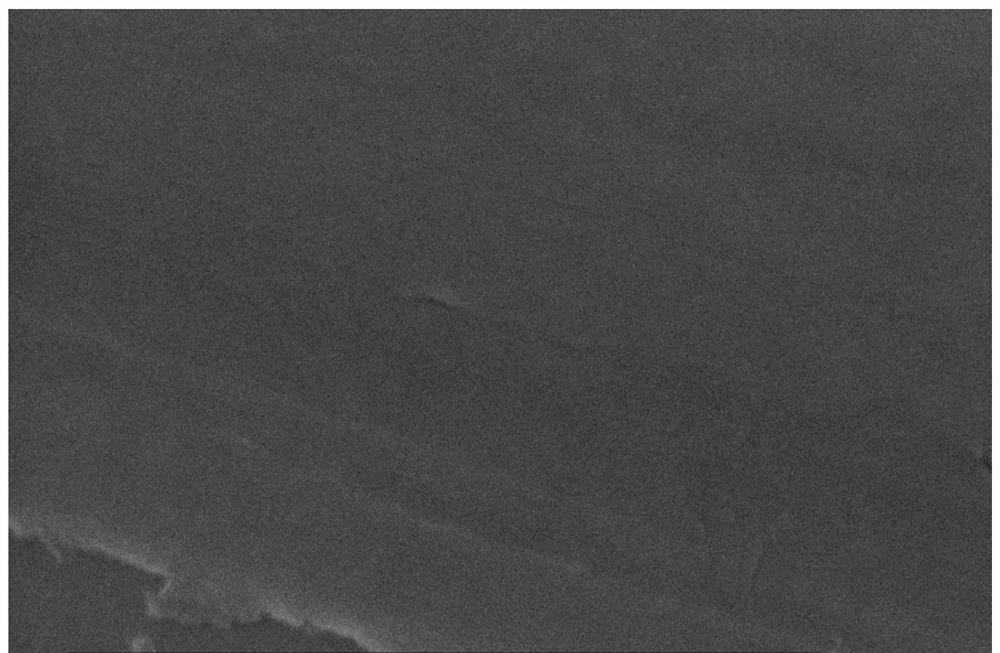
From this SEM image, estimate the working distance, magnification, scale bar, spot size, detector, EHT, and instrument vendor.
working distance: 5.3 mm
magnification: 100 K X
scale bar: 500 nm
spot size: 4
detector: TLD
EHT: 20 kV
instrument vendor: FEI